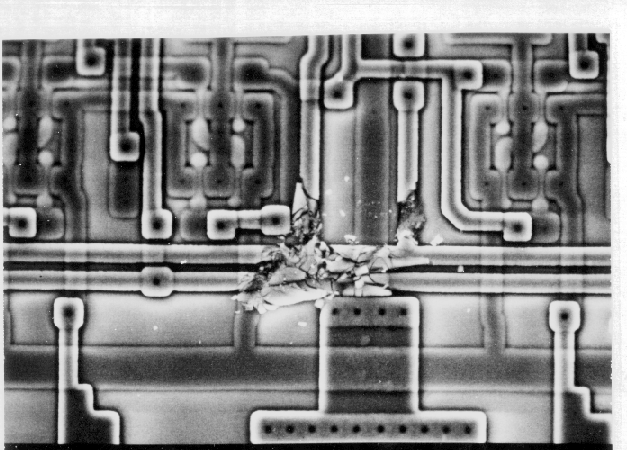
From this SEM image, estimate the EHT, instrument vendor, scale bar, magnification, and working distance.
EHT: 15 kV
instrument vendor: JEOL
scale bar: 10000 nm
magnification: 1 K X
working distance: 46 mm